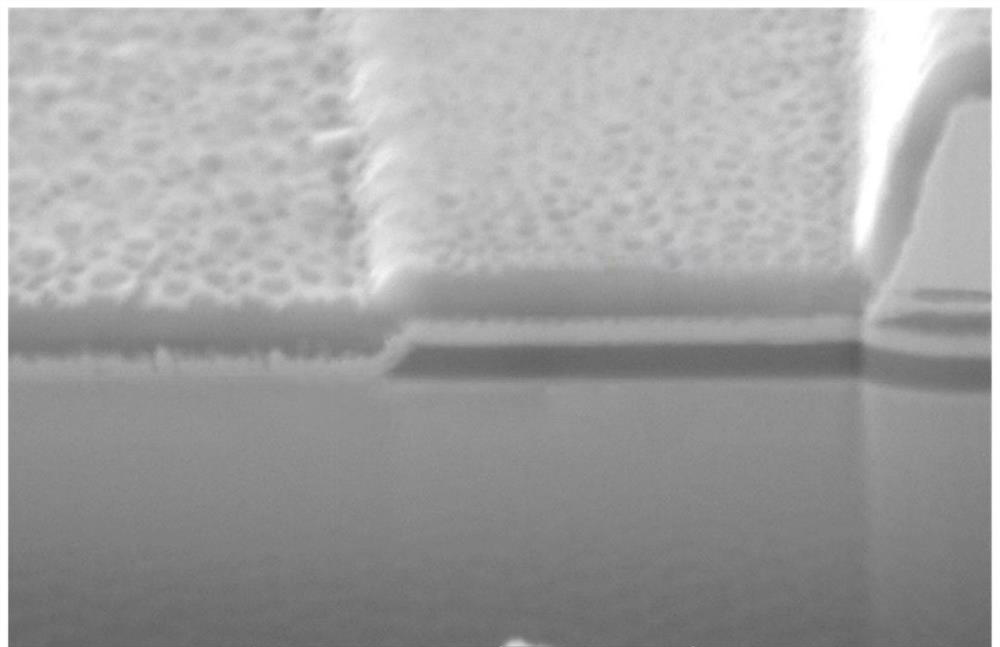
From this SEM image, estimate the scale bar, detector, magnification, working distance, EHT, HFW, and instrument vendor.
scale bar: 1000 nm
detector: SE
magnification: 40.6 K X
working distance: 9.04 mm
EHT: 5 kV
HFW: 5.12 µm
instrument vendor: Tescan